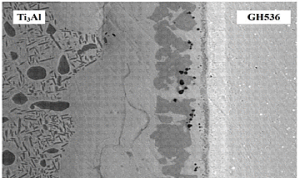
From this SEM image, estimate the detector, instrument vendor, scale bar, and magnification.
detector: BEI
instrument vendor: JEOL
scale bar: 20000 nm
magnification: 0.8 K X